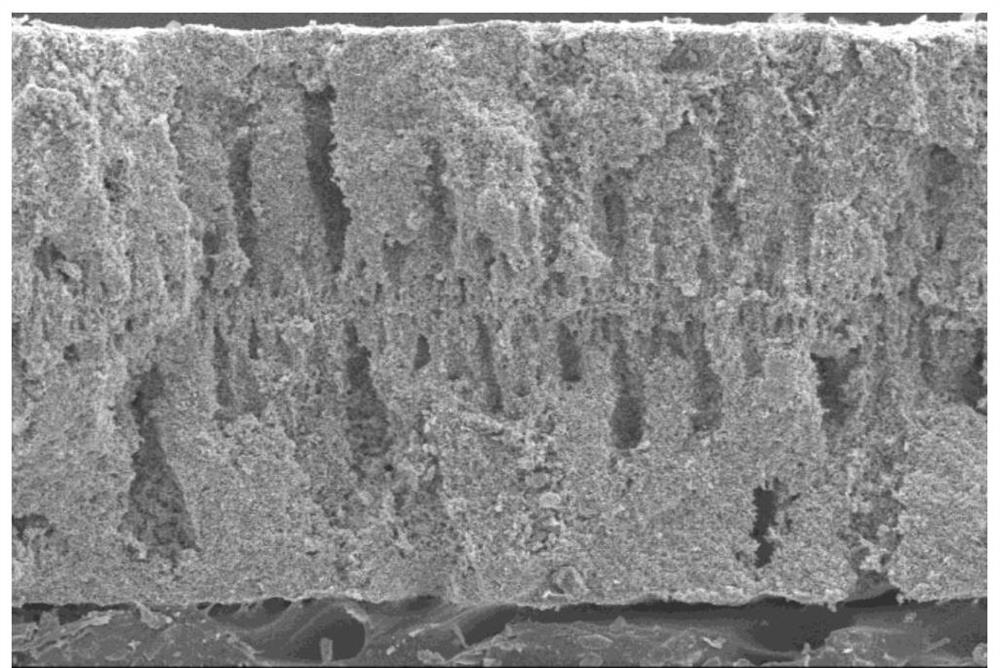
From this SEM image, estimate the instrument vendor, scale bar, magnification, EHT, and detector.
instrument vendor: JEOL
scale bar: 100000 nm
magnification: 0.11 K X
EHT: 20 kV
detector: SEI